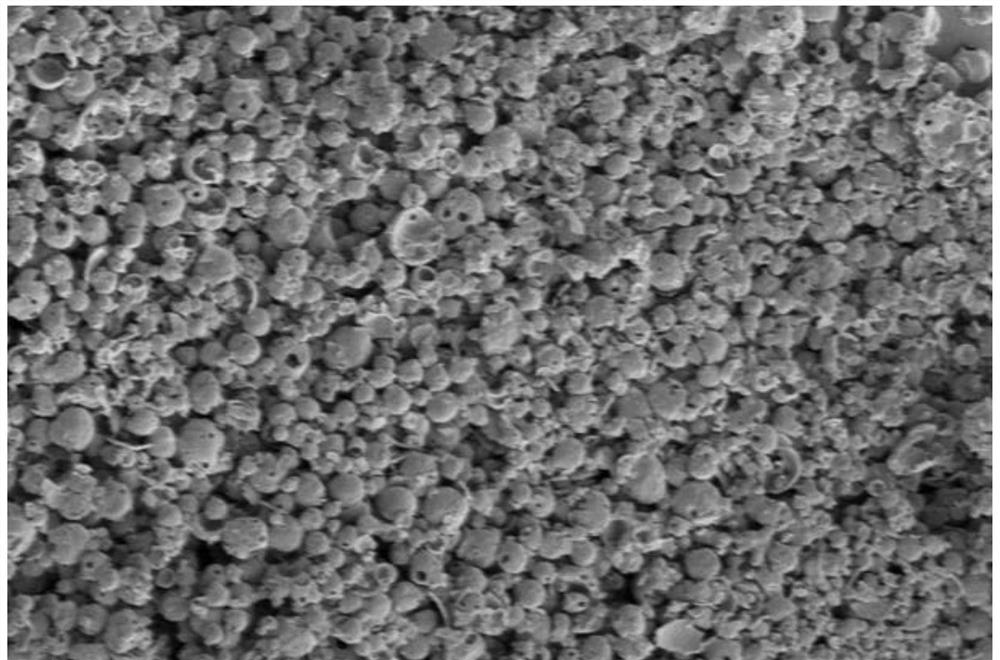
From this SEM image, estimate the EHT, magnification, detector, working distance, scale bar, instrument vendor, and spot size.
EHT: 5 kV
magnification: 5 K X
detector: ETD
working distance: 5.3 mm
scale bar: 40000 nm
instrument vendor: FEI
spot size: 3.5